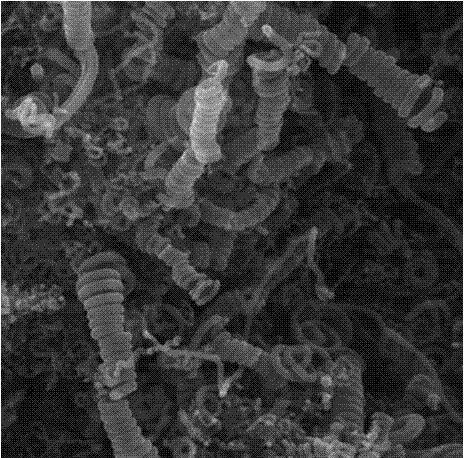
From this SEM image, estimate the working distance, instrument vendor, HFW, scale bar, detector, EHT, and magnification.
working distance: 7.62 mm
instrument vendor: Tescan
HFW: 11.9 µm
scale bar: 2000 nm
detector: SE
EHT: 20 kV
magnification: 12.5 K X